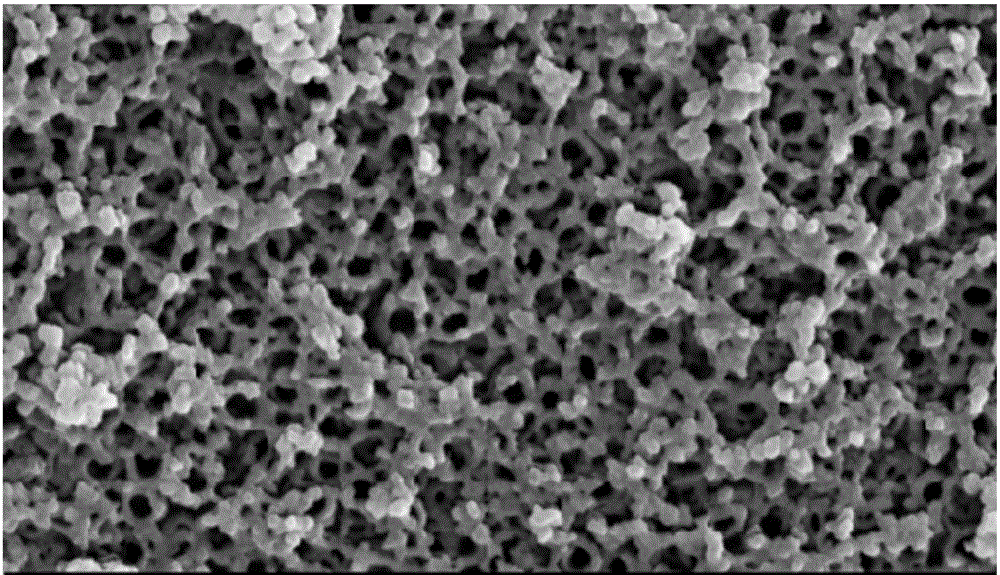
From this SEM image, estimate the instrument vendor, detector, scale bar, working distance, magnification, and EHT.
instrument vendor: Zeiss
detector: SE2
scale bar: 200 nm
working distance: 11.3 mm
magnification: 100 K X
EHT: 15 kV